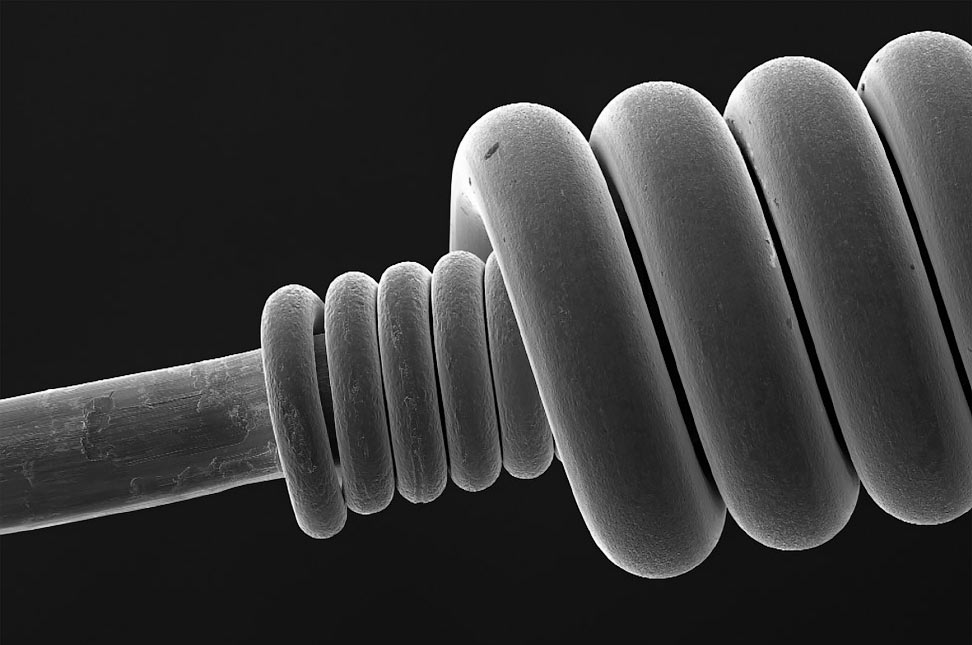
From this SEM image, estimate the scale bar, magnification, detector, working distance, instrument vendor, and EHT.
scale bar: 100000 nm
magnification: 0.035 K X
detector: SE2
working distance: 25.4 mm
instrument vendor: Zeiss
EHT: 20 kV